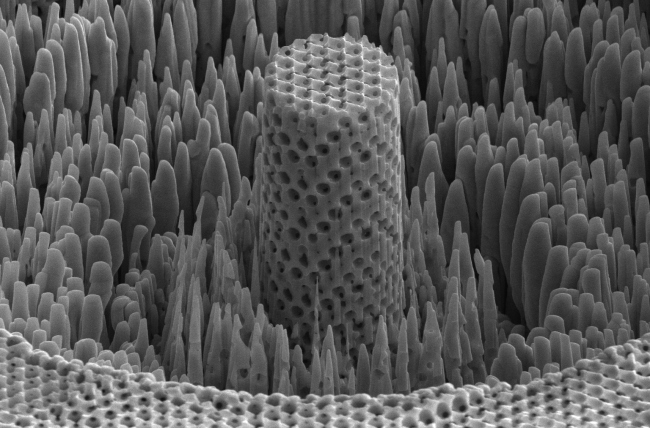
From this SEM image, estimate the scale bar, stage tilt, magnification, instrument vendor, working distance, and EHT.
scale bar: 5000 nm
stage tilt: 52°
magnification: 11.998 K X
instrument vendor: FEI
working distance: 4.1 mm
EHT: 10 kV